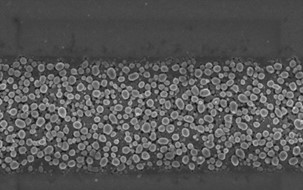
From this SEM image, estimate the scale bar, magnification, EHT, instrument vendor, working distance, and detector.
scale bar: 200 nm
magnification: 55.33 K X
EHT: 3 kV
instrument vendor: Zeiss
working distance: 4.5 mm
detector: InLens